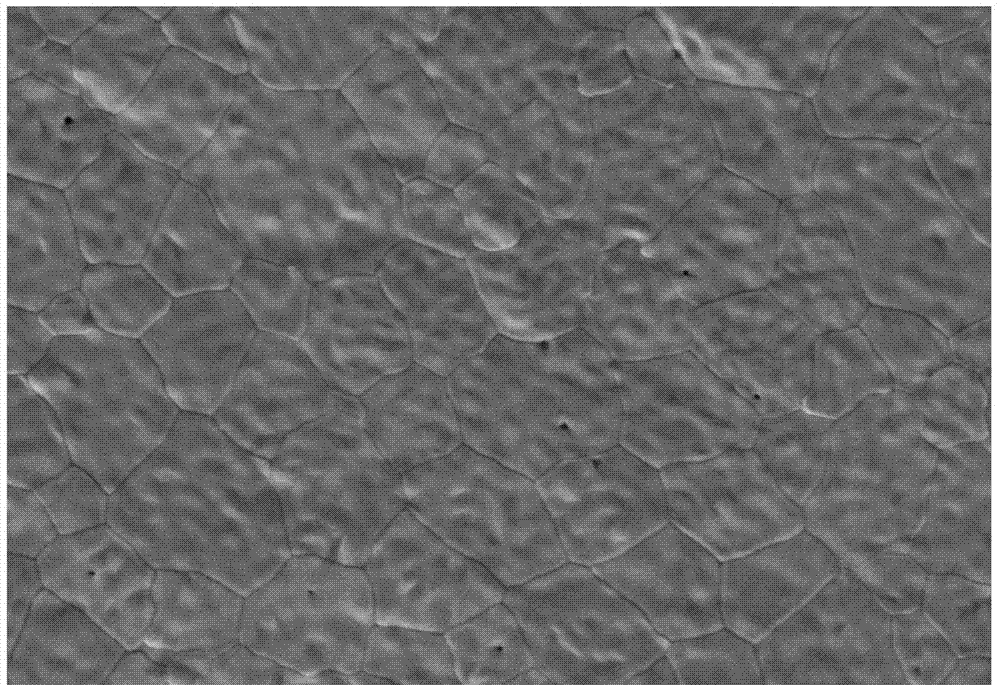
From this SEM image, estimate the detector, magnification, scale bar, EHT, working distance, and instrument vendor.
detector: SE2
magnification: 5 K X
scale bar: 1000 nm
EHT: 5 kV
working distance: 9.6 mm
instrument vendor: Zeiss